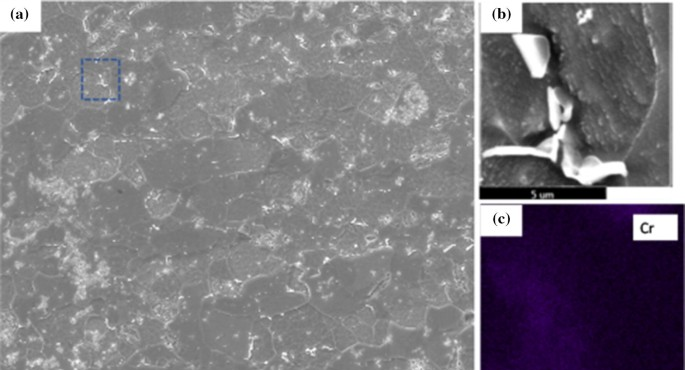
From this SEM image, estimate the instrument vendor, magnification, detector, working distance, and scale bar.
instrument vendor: FEI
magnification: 3 K X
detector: ETD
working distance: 15.3 mm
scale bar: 50000 nm